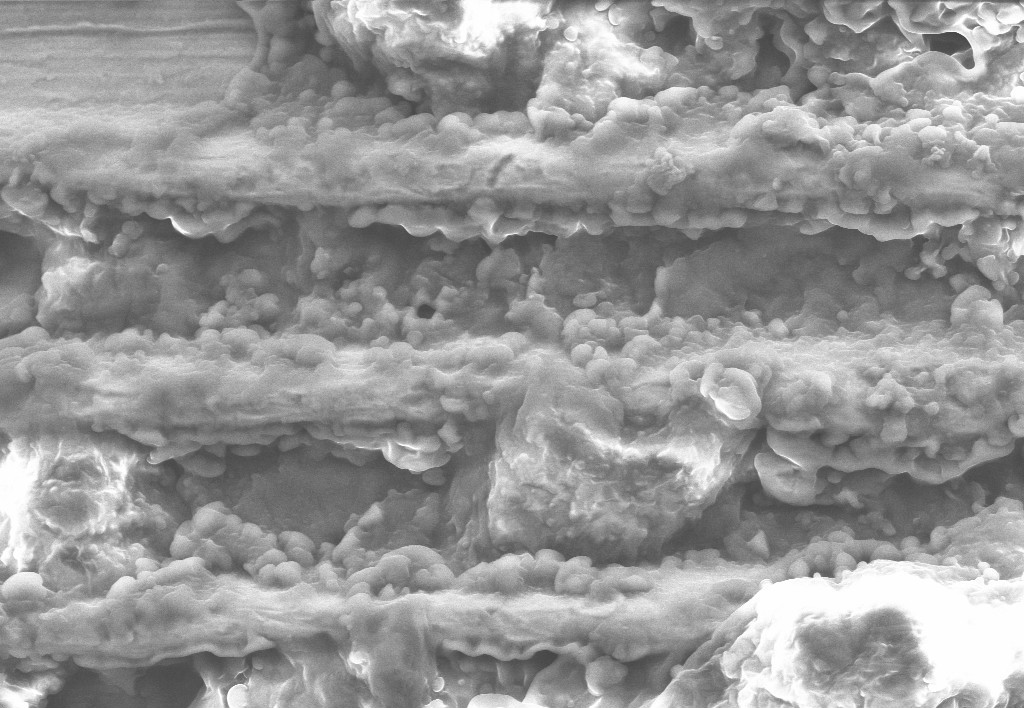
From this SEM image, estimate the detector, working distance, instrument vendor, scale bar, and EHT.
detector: SE1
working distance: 10 mm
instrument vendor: Zeiss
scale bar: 100000 nm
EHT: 20 kV